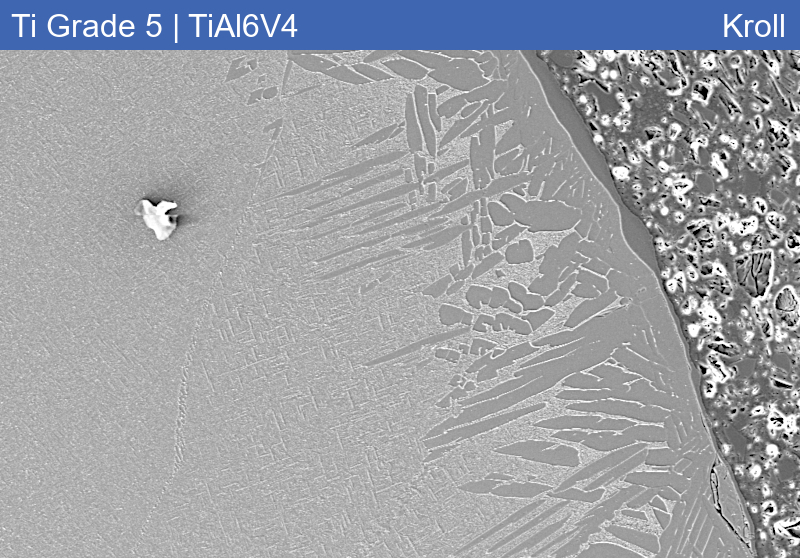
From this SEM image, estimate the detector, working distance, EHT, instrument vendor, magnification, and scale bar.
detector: SE2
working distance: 10.1 mm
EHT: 15 kV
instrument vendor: Zeiss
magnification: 0.2 K X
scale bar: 20000 nm